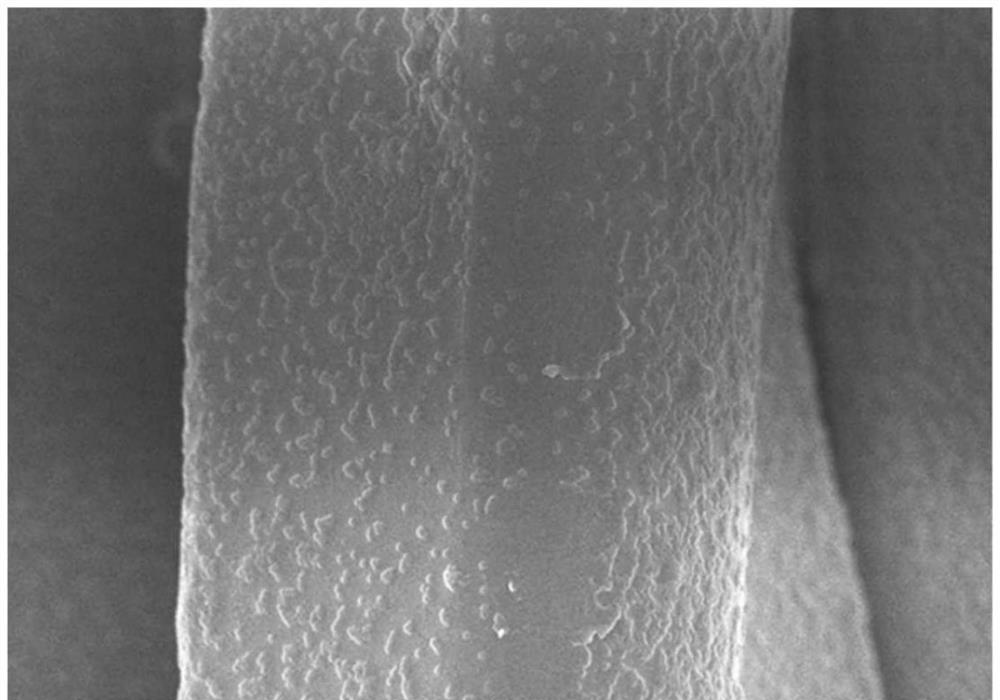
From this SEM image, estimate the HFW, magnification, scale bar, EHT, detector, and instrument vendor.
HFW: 24 µm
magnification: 5 K X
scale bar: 5000 nm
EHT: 3.5 kV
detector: SE A+B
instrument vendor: Hitachi